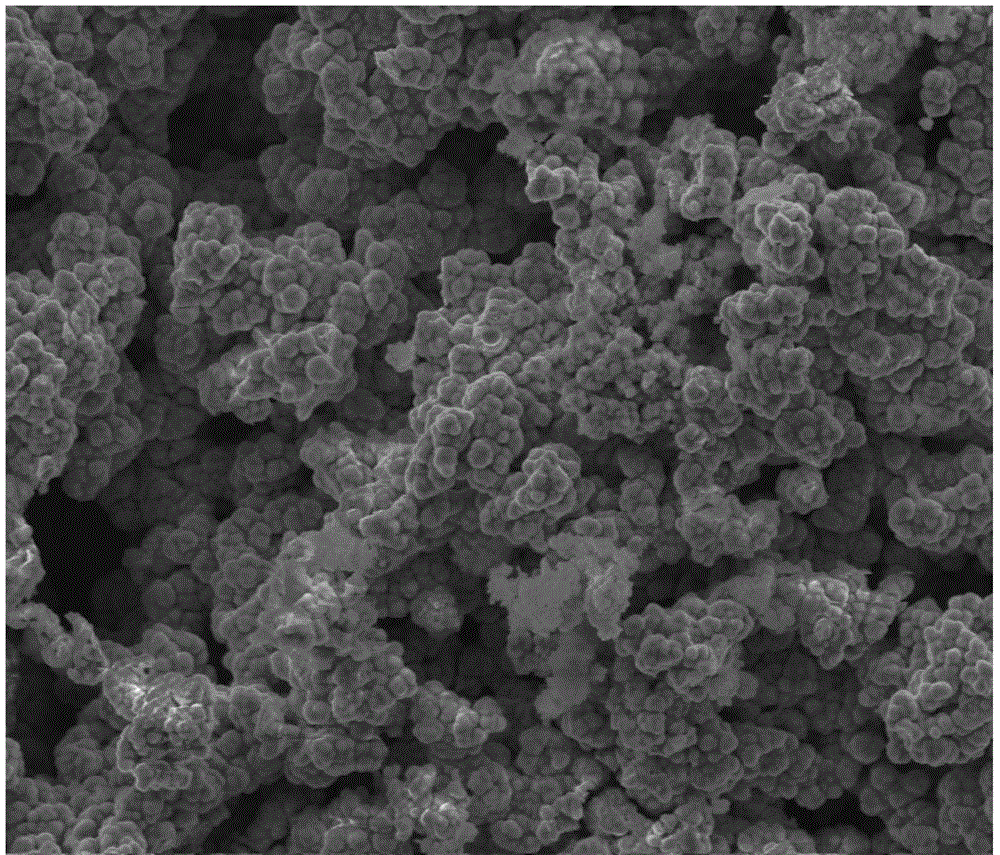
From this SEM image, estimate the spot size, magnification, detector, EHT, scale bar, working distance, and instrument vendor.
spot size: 3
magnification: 5 K X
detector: ETD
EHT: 10 kV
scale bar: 10000 nm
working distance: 7.4 mm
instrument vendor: FEI